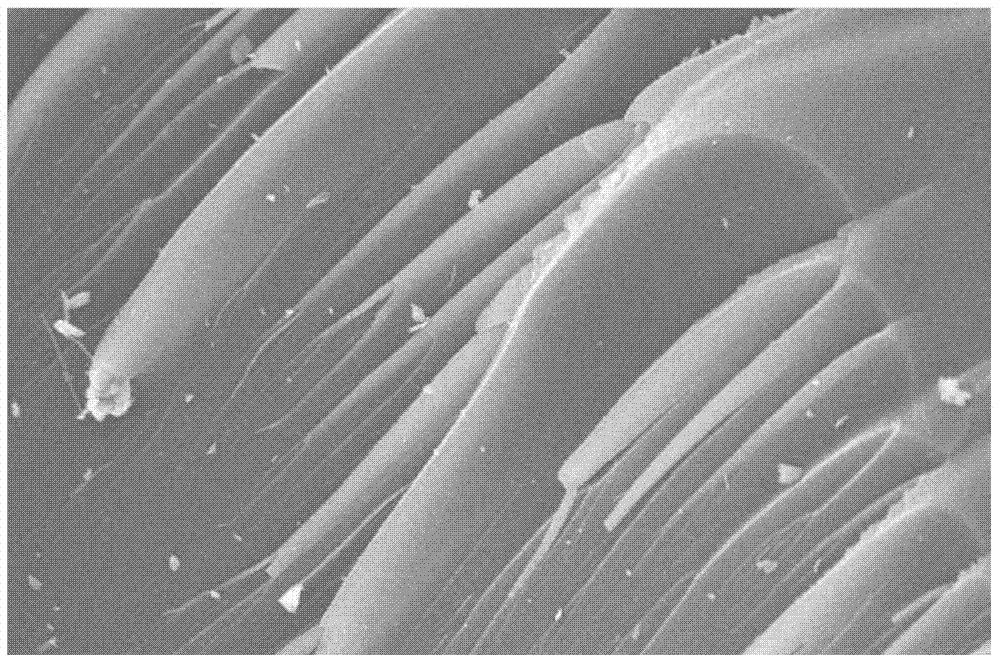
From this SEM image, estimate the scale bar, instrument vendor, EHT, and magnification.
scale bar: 10000 nm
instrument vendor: JEOL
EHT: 20 kV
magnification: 1 K X